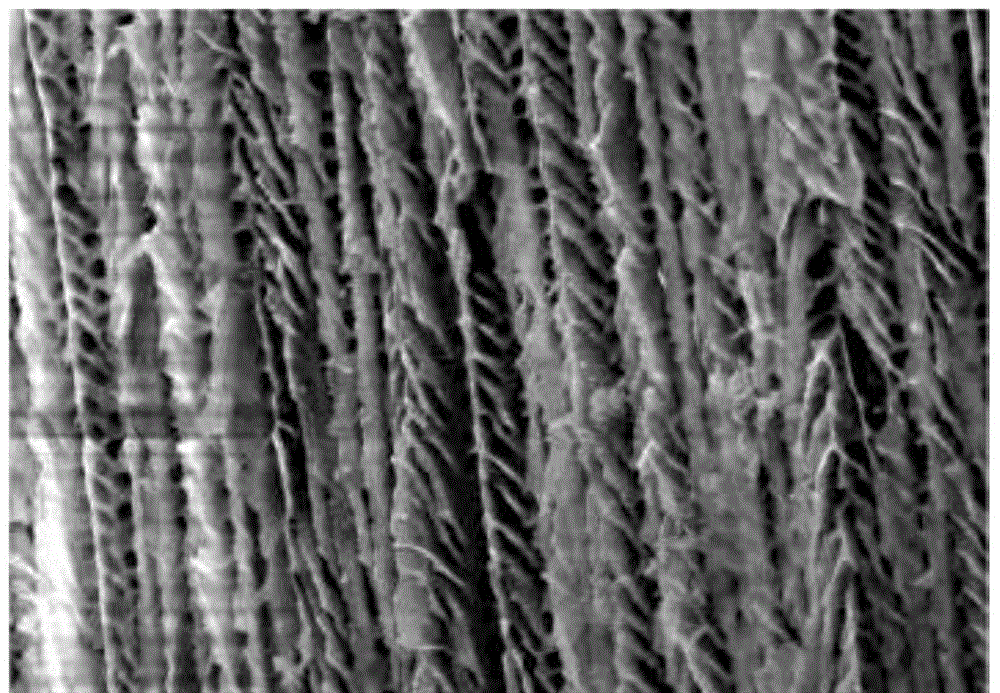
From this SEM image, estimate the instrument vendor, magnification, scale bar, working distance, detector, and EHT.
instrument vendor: Hitachi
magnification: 1 K X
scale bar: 50000 nm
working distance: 15.1 mm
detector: SE(M)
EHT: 15 kV